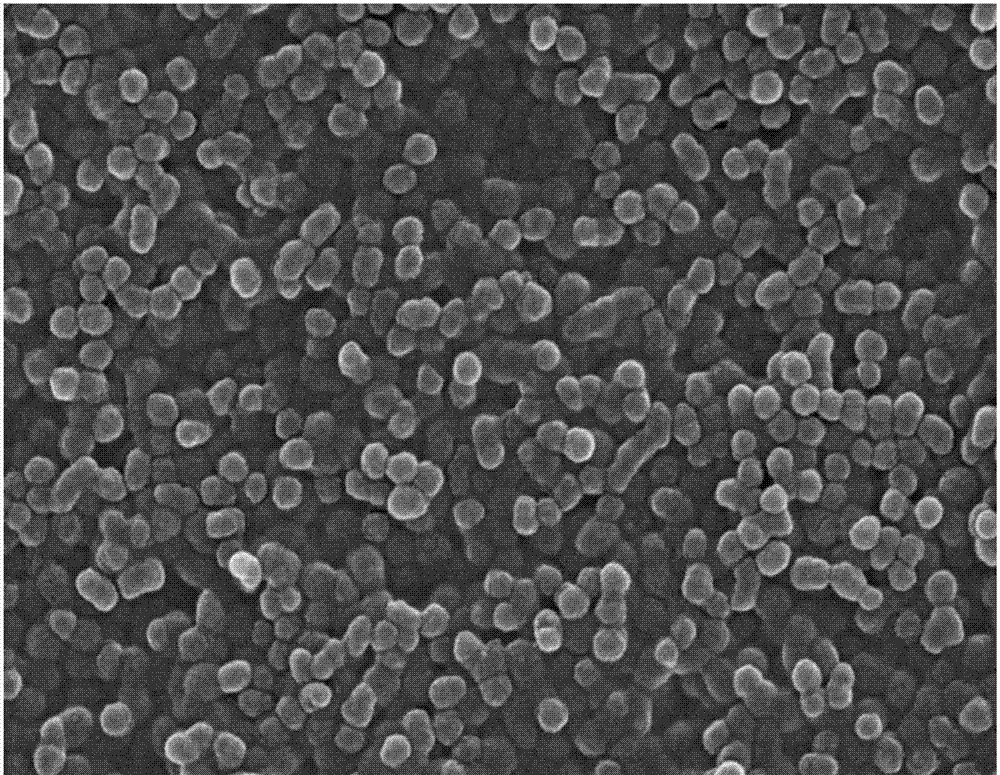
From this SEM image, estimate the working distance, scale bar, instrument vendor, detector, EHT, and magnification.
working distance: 9.2 mm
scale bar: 1000 nm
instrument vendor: Hitachi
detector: SE(UL)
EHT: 15 kV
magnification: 30 K X